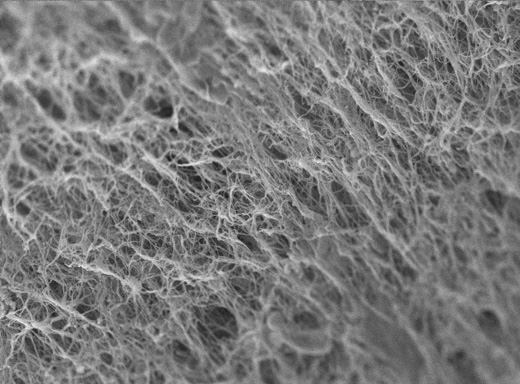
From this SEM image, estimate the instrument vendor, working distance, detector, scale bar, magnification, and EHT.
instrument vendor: JEOL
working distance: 6.9 mm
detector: SEI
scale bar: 1000 nm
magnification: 7.5 K X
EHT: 5 kV